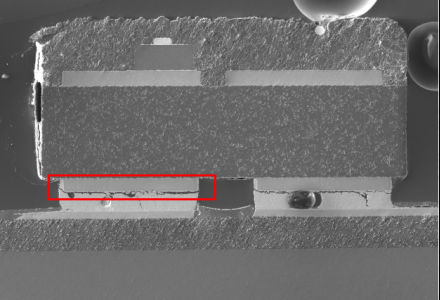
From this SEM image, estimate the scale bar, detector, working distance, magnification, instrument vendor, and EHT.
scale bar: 500000 nm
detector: LM(L)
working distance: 10.4 mm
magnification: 0.07 K X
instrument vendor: Hitachi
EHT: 15 kV